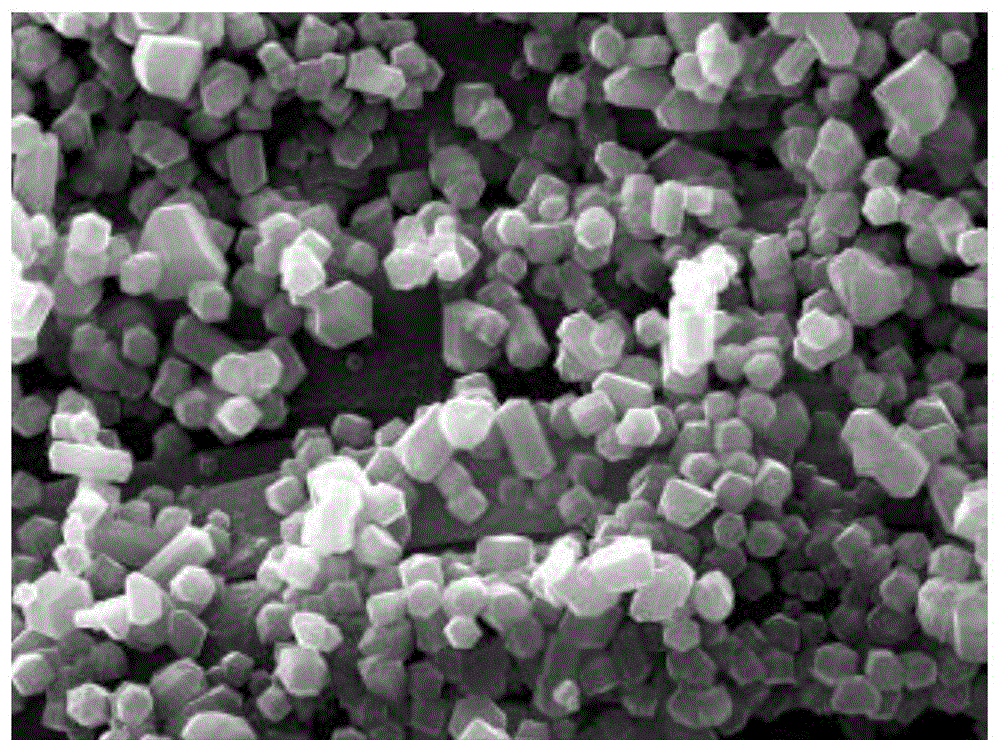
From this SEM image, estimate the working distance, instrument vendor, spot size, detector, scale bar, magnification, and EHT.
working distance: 12.6 mm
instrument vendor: JEOL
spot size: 30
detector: SED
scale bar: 10000 nm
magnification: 2.5 K X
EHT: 20 kV